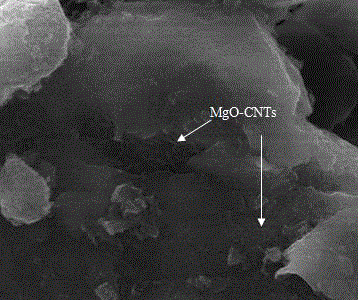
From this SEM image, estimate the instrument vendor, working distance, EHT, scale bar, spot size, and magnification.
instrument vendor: FEI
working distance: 7.3 mm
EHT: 20 kV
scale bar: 5000 nm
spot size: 3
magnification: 12 K X